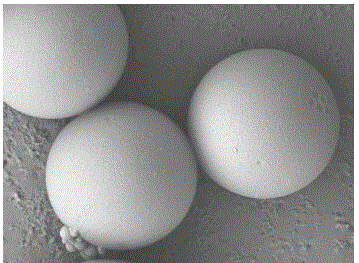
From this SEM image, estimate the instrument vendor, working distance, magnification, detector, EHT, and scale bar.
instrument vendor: JEOL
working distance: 7.9 mm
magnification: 5 K X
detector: LEI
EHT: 5 kV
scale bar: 1000 nm